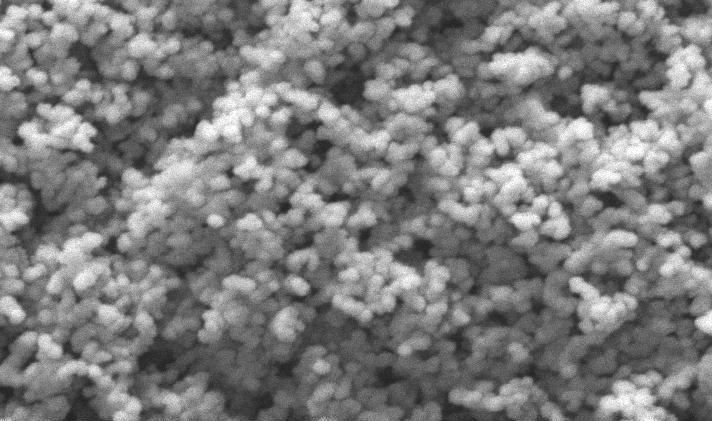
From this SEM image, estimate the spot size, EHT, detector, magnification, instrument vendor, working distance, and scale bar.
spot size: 3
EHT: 20 kV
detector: SE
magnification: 64 K X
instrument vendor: FEI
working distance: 10.2 mm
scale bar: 1000 nm